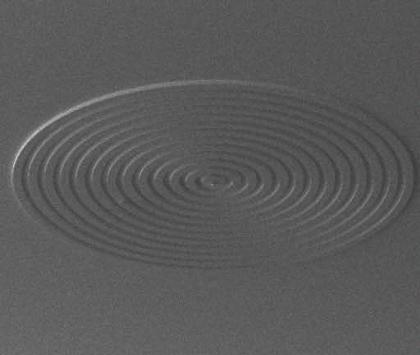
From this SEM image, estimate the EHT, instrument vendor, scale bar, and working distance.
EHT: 10 kV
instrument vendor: FEI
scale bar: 1000000 nm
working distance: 15.2 mm